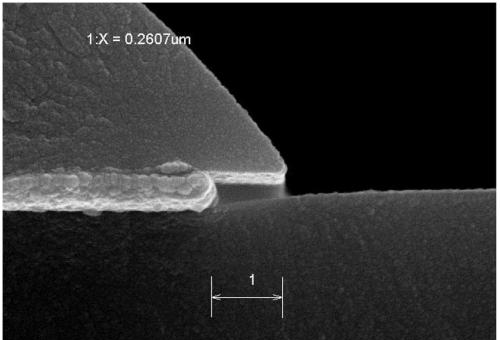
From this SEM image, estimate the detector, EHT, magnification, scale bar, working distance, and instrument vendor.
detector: SE(M)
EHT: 15 kV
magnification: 70 K X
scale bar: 500 nm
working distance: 7.5 mm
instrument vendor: Hitachi